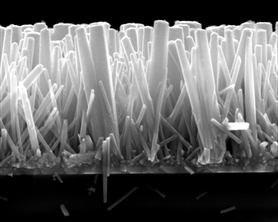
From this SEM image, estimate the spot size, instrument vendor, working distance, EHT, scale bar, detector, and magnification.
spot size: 2.5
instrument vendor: FEI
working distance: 9.2 mm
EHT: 10 kV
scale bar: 1000 nm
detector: ETD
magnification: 80 K X